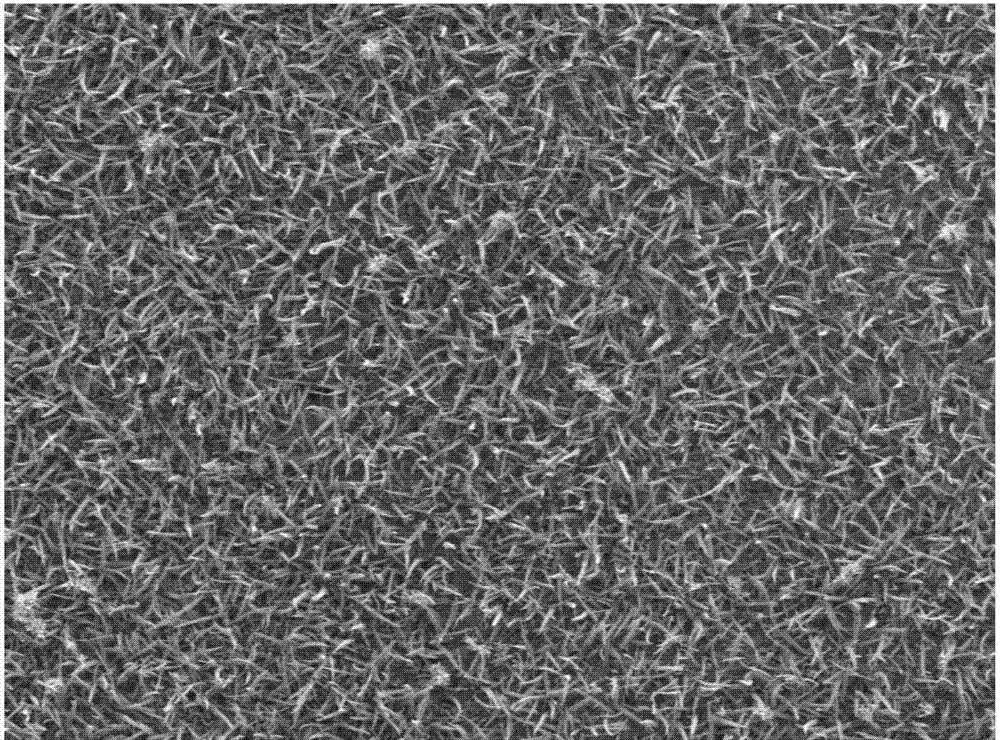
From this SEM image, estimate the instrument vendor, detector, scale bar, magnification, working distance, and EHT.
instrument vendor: JEOL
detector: LED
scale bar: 10000 nm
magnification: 2 K X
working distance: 8.1 mm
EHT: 3 kV